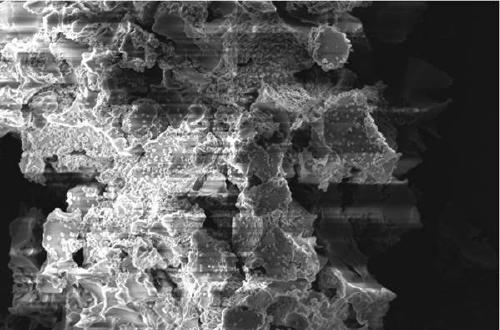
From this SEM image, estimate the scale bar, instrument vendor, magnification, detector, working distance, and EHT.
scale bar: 2000 nm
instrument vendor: Zeiss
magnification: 3 K X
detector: SE2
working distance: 8.9 mm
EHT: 2.5 kV